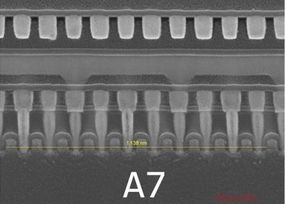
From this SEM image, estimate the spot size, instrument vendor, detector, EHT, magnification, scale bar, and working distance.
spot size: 3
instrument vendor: FEI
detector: TLD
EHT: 10 kV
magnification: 100 K X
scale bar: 200 nm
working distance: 5.2 mm